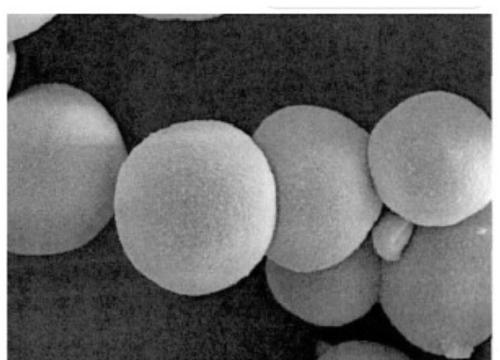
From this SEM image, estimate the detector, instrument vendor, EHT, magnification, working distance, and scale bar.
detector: SE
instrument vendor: Hitachi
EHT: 15 kV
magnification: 3 K X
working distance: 5.3 mm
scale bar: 10000 nm